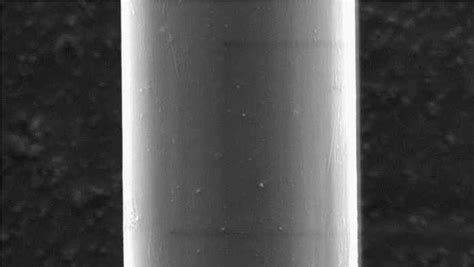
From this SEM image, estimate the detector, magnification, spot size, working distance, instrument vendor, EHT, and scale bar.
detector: SE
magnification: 5 K X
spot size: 3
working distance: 9.7 mm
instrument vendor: FEI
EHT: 5 kV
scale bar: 5000000 nm